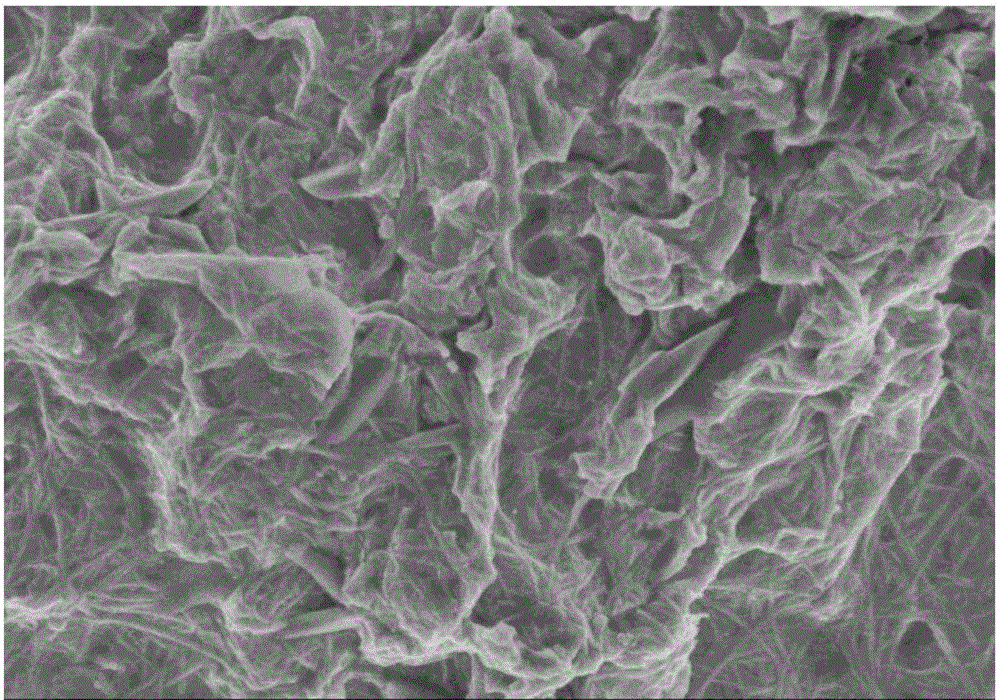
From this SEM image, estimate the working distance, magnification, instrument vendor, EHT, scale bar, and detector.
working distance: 8.6 mm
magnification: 22 K X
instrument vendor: Hitachi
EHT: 3 kV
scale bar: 2000 nm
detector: SE(M)